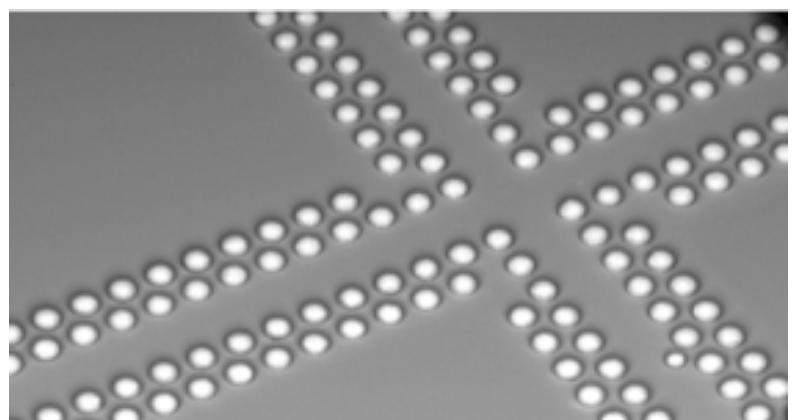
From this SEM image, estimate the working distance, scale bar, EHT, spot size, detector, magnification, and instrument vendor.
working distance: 16.2 mm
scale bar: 1000000 nm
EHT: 20 kV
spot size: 3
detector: BSE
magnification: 0.053 K X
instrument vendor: FEI